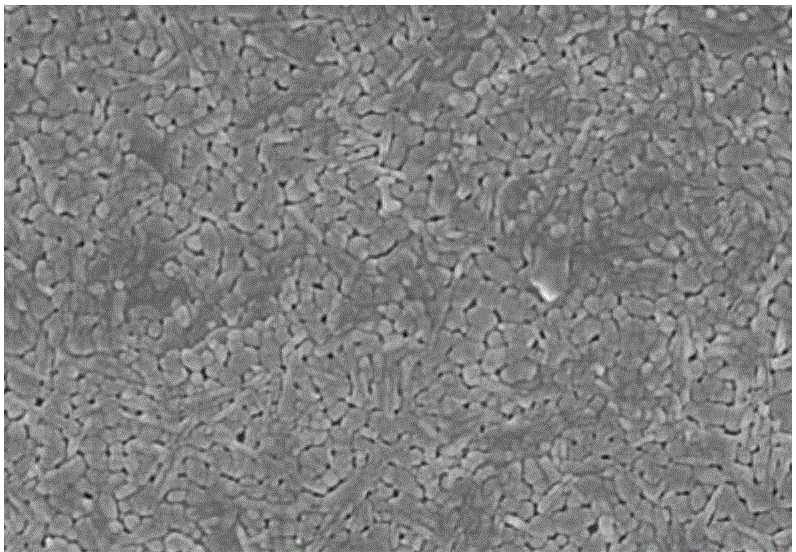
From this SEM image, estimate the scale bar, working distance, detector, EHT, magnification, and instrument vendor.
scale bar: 1000 nm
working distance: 4.4 mm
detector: SE(M)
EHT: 5 kV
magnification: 30 K X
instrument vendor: Hitachi